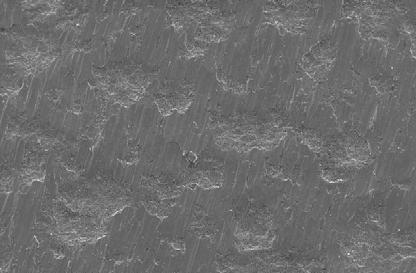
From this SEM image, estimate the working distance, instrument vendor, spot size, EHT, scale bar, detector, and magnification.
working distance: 11.2 mm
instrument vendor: FEI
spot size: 4.4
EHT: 20 kV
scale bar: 200000 nm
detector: SE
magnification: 0.2 K X